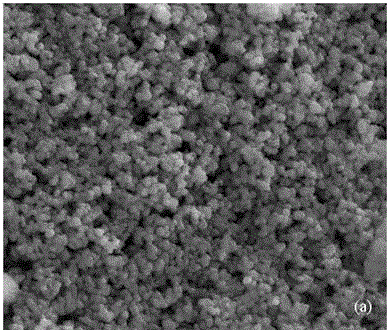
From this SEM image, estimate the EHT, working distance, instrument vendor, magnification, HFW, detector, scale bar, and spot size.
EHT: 15 kV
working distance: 8.6 mm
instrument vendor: FEI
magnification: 15 K X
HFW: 9.95 µm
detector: ETD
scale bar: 3000 nm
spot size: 3.5